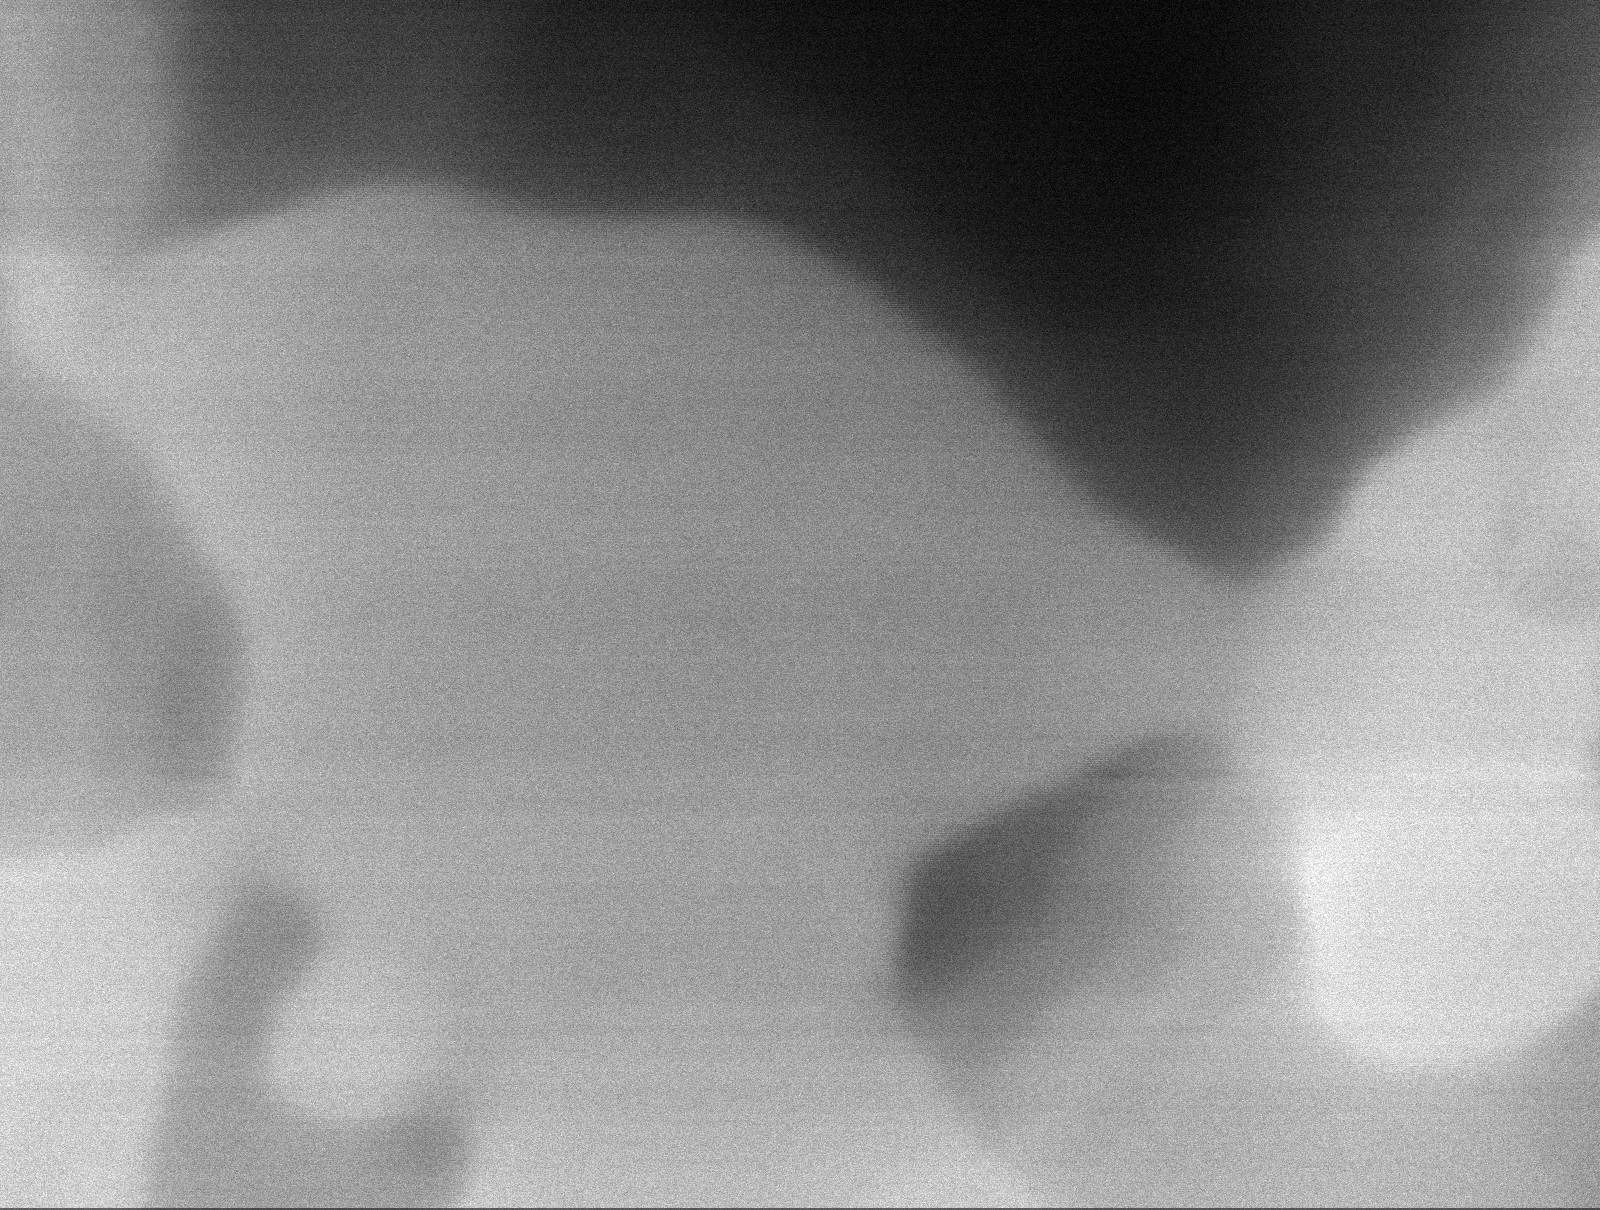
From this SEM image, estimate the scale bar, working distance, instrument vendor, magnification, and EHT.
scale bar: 60 nm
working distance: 6 mm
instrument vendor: Tescan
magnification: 370 K X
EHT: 12 kV